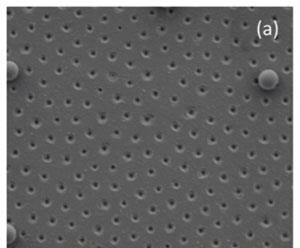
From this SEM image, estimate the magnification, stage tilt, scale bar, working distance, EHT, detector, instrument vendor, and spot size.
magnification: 40 K X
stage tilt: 0°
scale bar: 2000 nm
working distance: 8 mm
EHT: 2 kV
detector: ETD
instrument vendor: FEI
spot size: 3.5